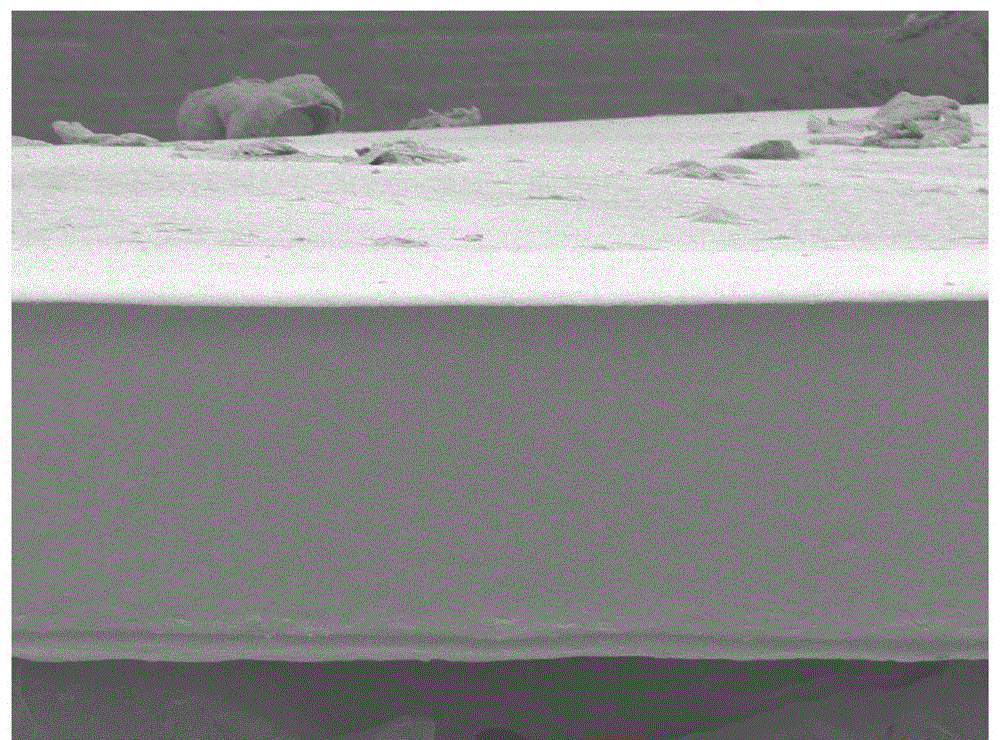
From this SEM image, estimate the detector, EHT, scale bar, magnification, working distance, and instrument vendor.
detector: SEI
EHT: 5 kV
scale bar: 10000 nm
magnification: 0.37 K X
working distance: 8 mm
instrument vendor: JEOL